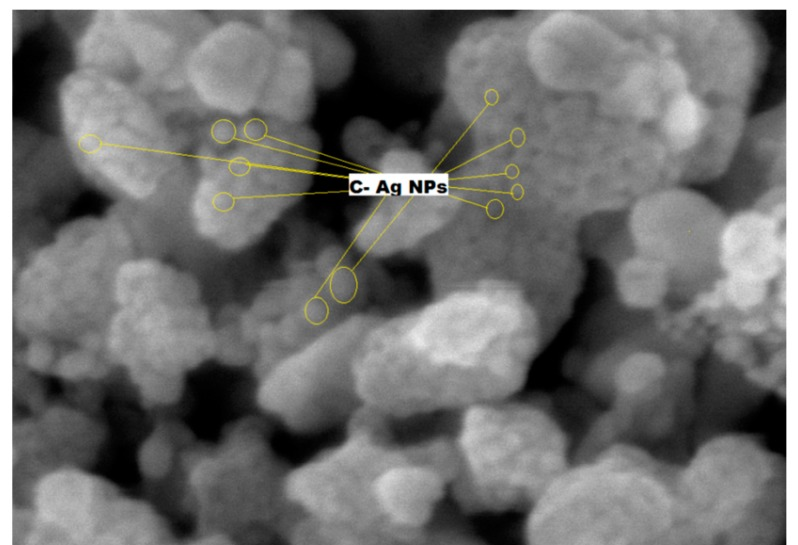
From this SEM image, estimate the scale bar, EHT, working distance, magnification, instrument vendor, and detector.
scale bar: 100 nm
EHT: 5 kV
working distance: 3.1 mm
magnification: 200 K X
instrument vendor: JEOL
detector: SEI